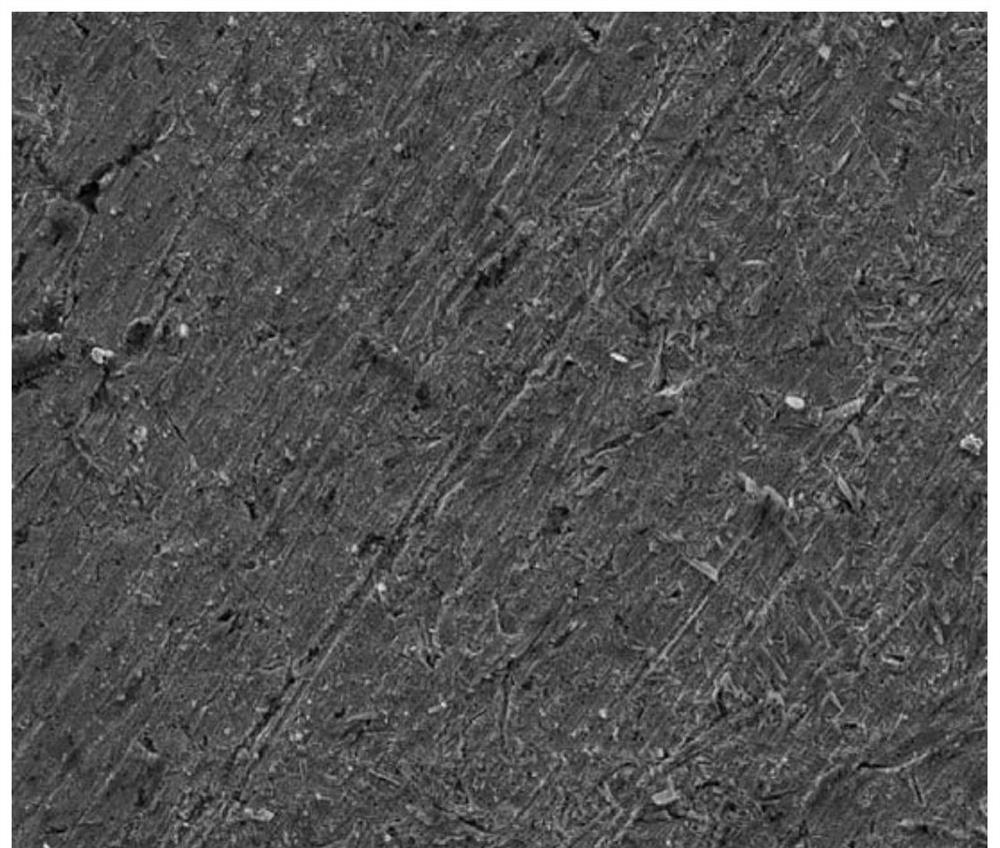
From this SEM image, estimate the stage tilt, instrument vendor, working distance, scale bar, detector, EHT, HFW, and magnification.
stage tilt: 0°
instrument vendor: FEI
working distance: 11.4 mm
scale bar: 100000 nm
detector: ETD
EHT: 20 kV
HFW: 298 µm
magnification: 1 K X